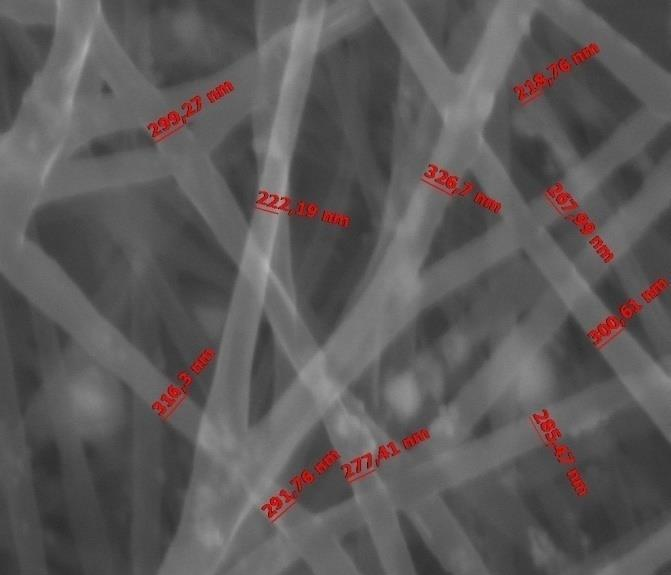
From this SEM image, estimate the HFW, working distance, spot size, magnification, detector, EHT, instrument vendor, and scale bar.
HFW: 5.97 µm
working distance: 5.8 mm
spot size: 5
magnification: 50 K X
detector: LVD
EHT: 18 kV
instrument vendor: FEI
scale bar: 2000 nm